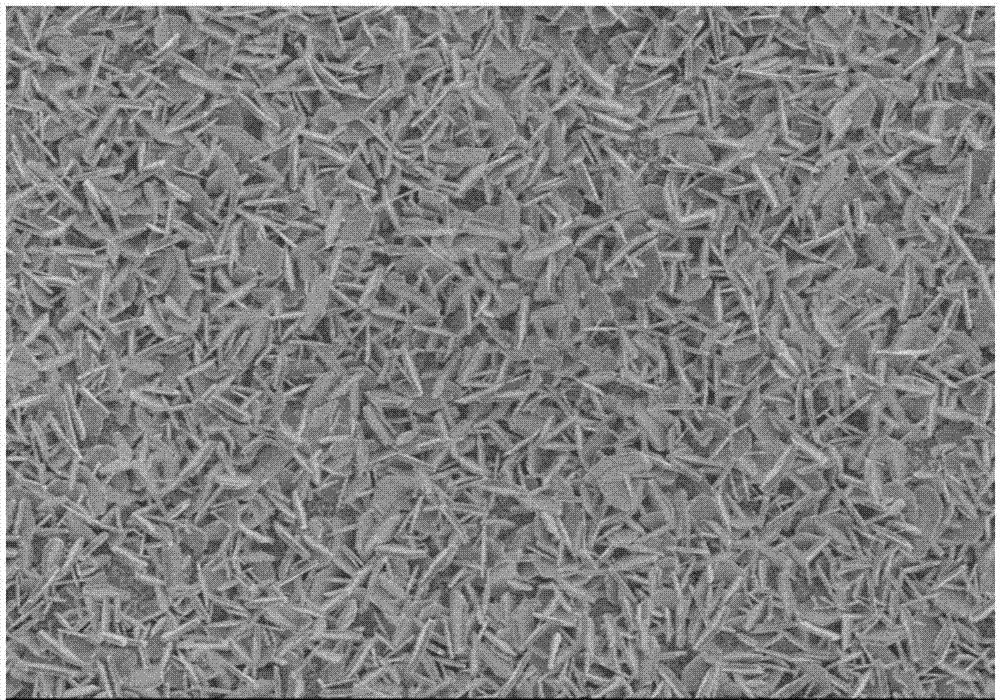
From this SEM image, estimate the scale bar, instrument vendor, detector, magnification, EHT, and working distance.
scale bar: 10000 nm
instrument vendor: Hitachi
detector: SE(M)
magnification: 5 K X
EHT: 3 kV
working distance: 8.7 mm